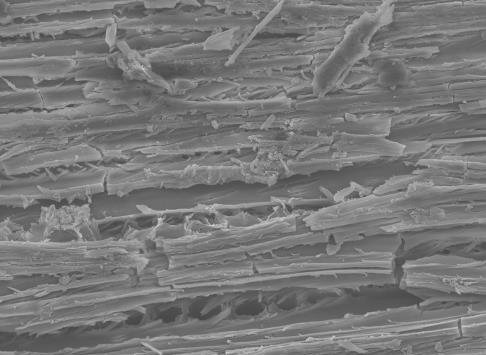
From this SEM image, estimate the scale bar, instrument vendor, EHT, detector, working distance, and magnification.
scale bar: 10000 nm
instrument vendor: JEOL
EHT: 15 kV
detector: SEI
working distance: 19 mm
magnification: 0.5 K X